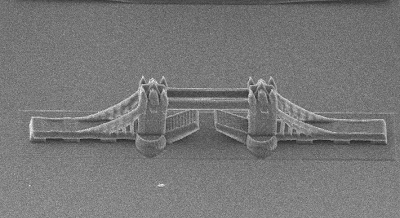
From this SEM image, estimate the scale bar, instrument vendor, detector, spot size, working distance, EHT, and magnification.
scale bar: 100000 nm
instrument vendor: FEI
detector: SE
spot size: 4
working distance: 27.1 mm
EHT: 12 kV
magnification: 0.368 K X